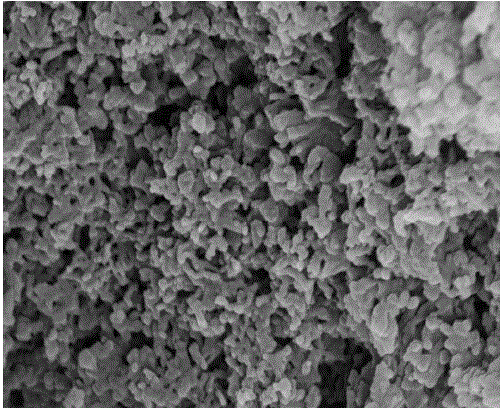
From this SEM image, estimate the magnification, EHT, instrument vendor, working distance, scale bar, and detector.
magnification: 50 K X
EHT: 15 kV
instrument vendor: Hitachi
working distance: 7.5 mm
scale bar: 1000 nm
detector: SE(U)